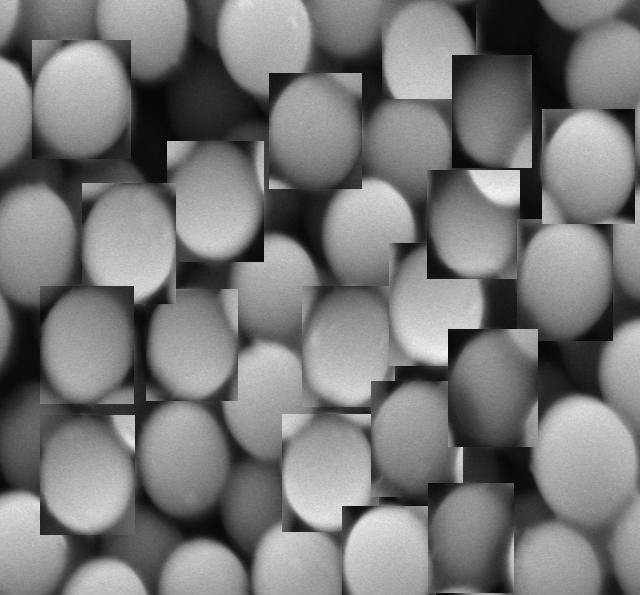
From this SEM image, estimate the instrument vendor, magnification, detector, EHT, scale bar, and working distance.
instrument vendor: JEOL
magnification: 50 K X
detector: LED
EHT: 5 kV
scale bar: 100 nm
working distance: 7.8 mm